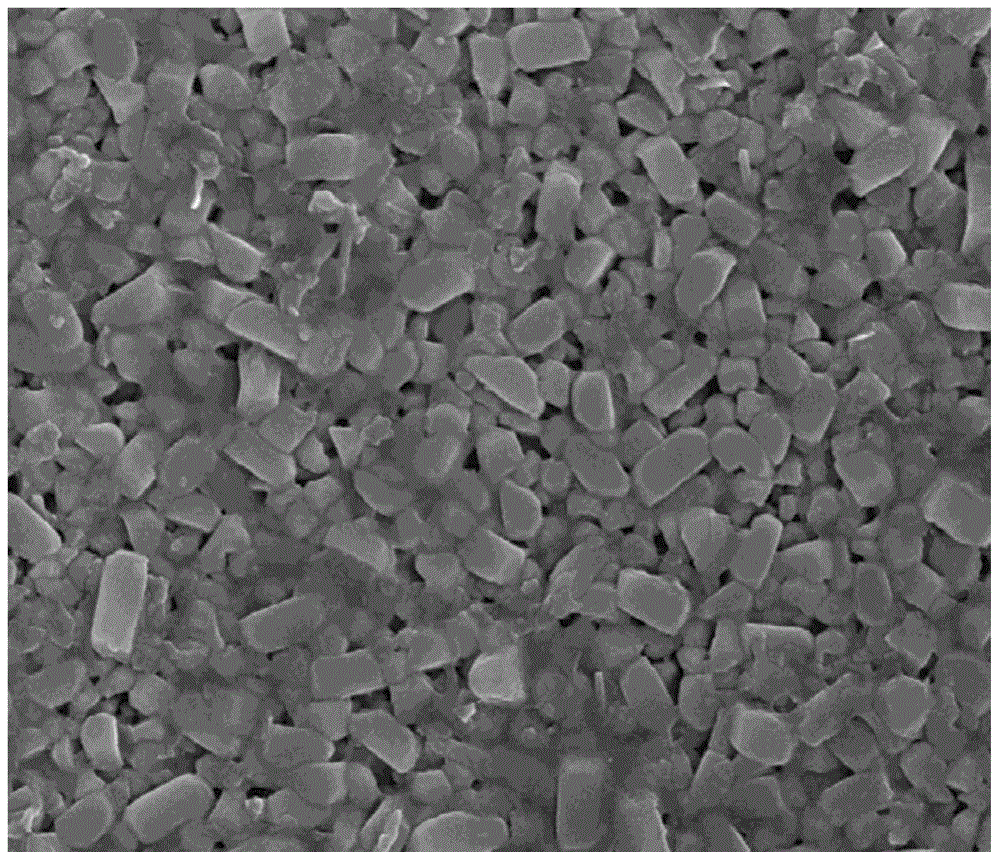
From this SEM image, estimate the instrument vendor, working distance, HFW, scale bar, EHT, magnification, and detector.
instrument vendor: FEI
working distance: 5.5 mm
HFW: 4.8 µm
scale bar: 1000 nm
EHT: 10 kV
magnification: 50 K X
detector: TLD